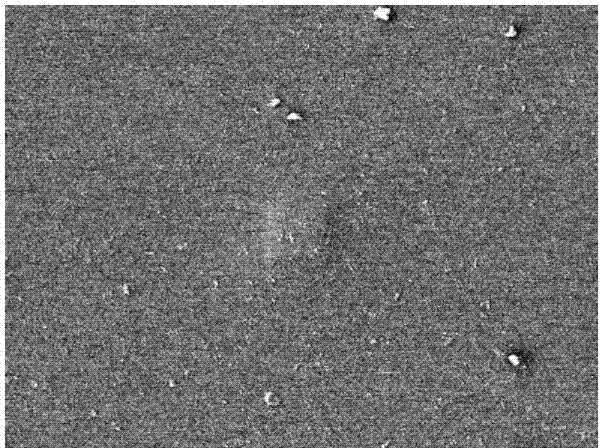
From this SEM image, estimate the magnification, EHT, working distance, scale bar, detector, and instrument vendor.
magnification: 5 K X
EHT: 20 kV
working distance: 14.9 mm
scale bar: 1000 nm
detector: LEI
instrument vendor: JEOL